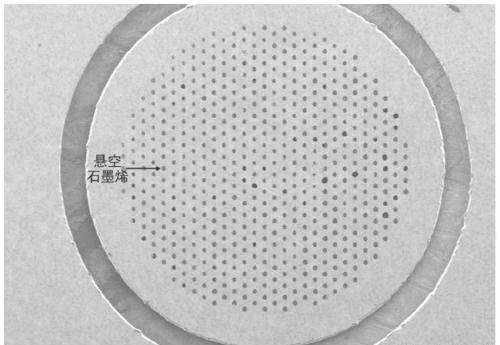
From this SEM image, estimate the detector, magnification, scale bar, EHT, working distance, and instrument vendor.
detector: SE(M)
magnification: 0.03 K X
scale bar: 1000000 nm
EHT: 1 kV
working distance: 9.8 mm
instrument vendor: Hitachi